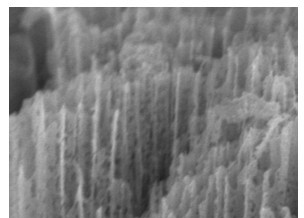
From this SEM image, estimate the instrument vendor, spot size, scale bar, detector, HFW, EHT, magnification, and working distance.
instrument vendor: FEI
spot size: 3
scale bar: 200 nm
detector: TLD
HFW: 1.9 µm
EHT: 5 kV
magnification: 150 K X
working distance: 4.3 mm